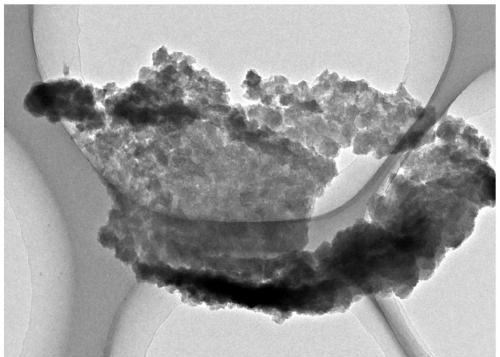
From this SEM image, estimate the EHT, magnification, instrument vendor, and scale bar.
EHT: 200 kV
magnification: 8 K X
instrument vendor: JEOL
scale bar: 500 nm